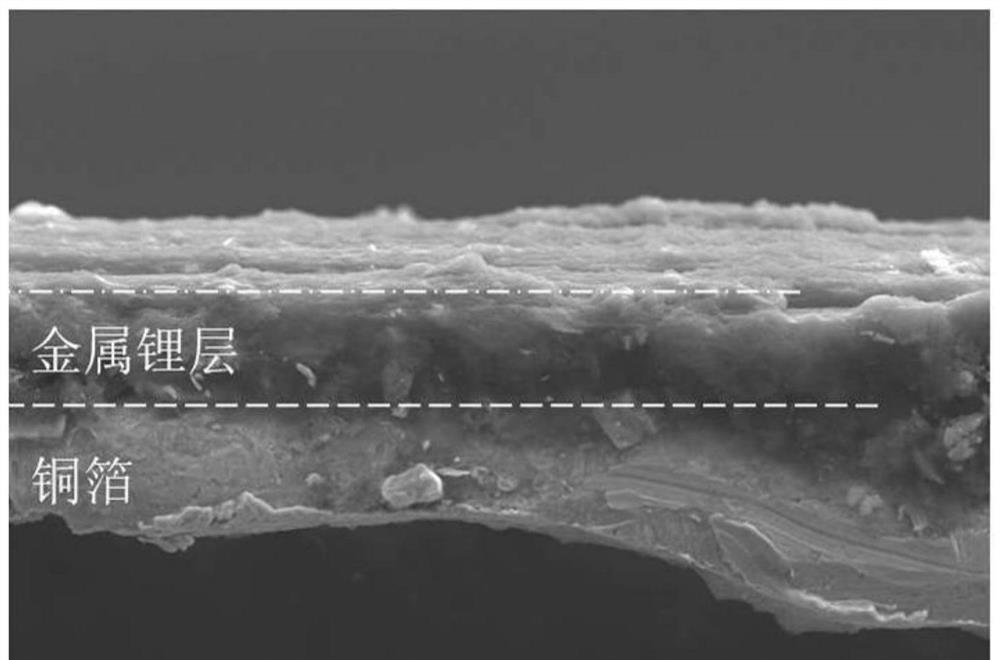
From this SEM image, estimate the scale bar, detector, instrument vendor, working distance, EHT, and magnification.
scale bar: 10000 nm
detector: SE2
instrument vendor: Zeiss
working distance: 6.8 mm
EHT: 15 kV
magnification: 1.15 K X